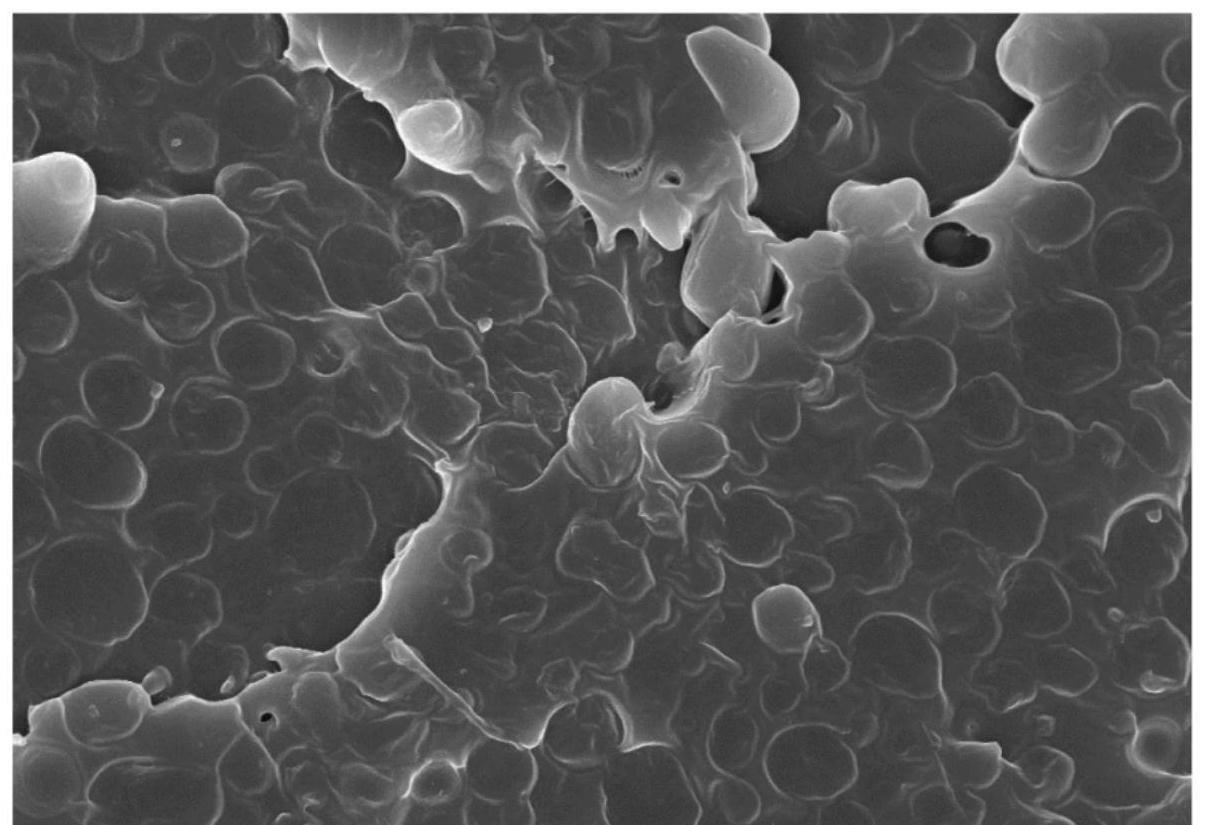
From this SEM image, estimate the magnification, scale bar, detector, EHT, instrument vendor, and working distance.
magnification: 5 K X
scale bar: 10000 nm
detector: SE(U)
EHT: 10 kV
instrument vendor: Hitachi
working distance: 15.4 mm